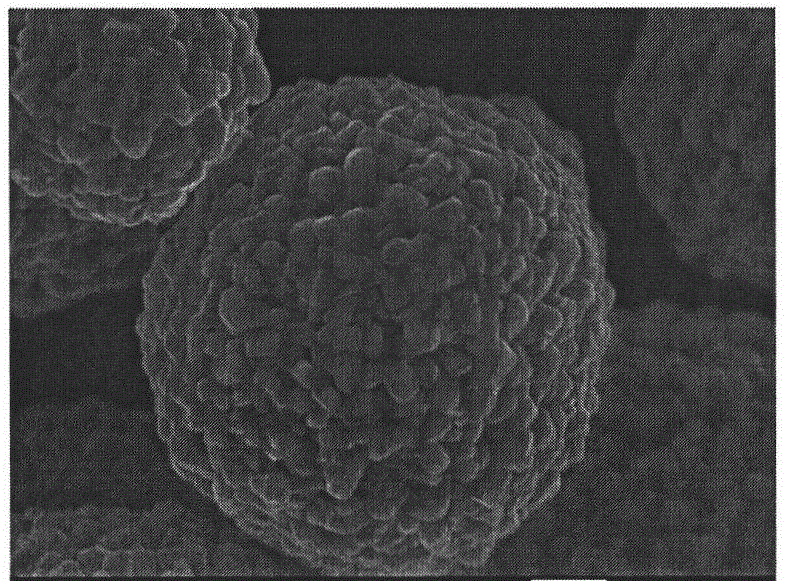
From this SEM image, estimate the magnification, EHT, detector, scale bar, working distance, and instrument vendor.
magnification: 12 K X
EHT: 10 kV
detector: SEI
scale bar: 1000 nm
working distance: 7.6 mm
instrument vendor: JEOL